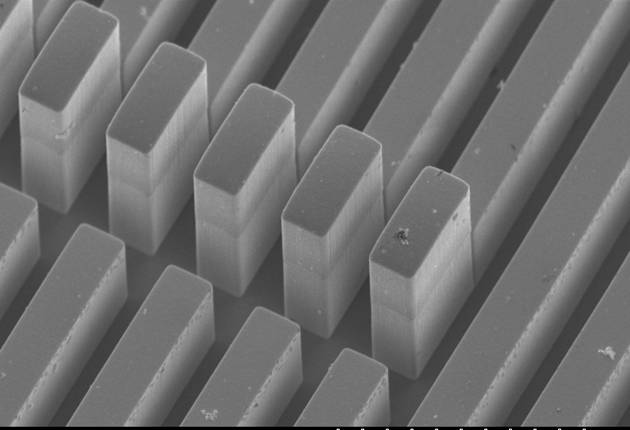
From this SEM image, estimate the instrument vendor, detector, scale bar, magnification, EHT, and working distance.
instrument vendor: Hitachi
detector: SE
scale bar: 50000 nm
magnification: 1 K X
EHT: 5 kV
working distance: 17.7 mm